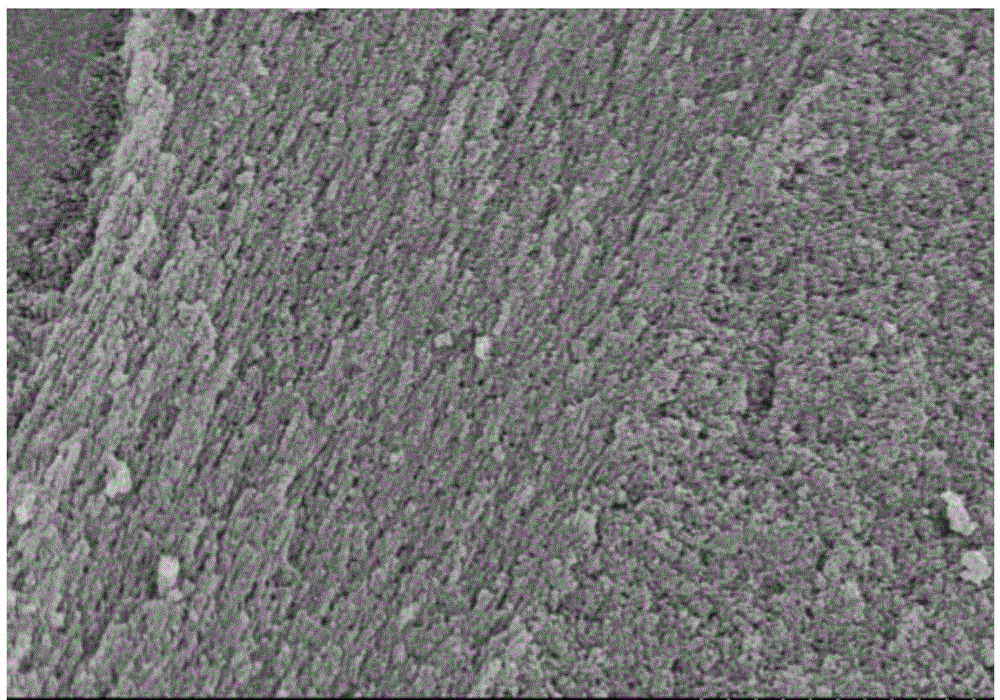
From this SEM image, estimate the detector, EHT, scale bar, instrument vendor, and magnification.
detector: SE(M)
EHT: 5 kV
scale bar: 1000 nm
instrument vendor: Hitachi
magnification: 35 K X